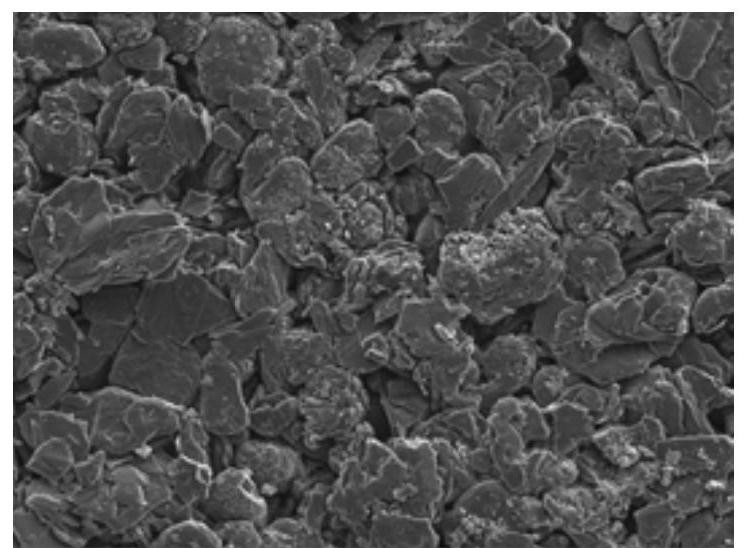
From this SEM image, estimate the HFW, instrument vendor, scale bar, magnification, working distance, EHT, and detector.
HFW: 138 µm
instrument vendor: Tescan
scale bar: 20000 nm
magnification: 2 K X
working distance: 5.03 mm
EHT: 5 kV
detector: SE